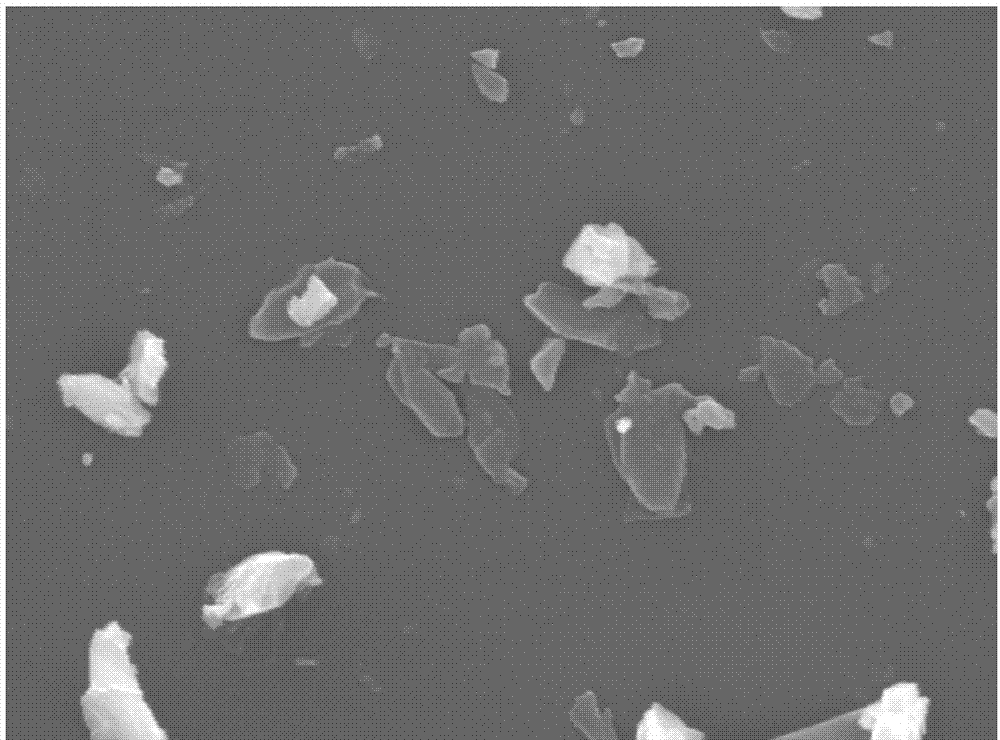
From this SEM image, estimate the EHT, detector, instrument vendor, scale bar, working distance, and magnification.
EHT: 10 kV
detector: LED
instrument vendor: JEOL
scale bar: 1000 nm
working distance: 10.3 mm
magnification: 18 K X